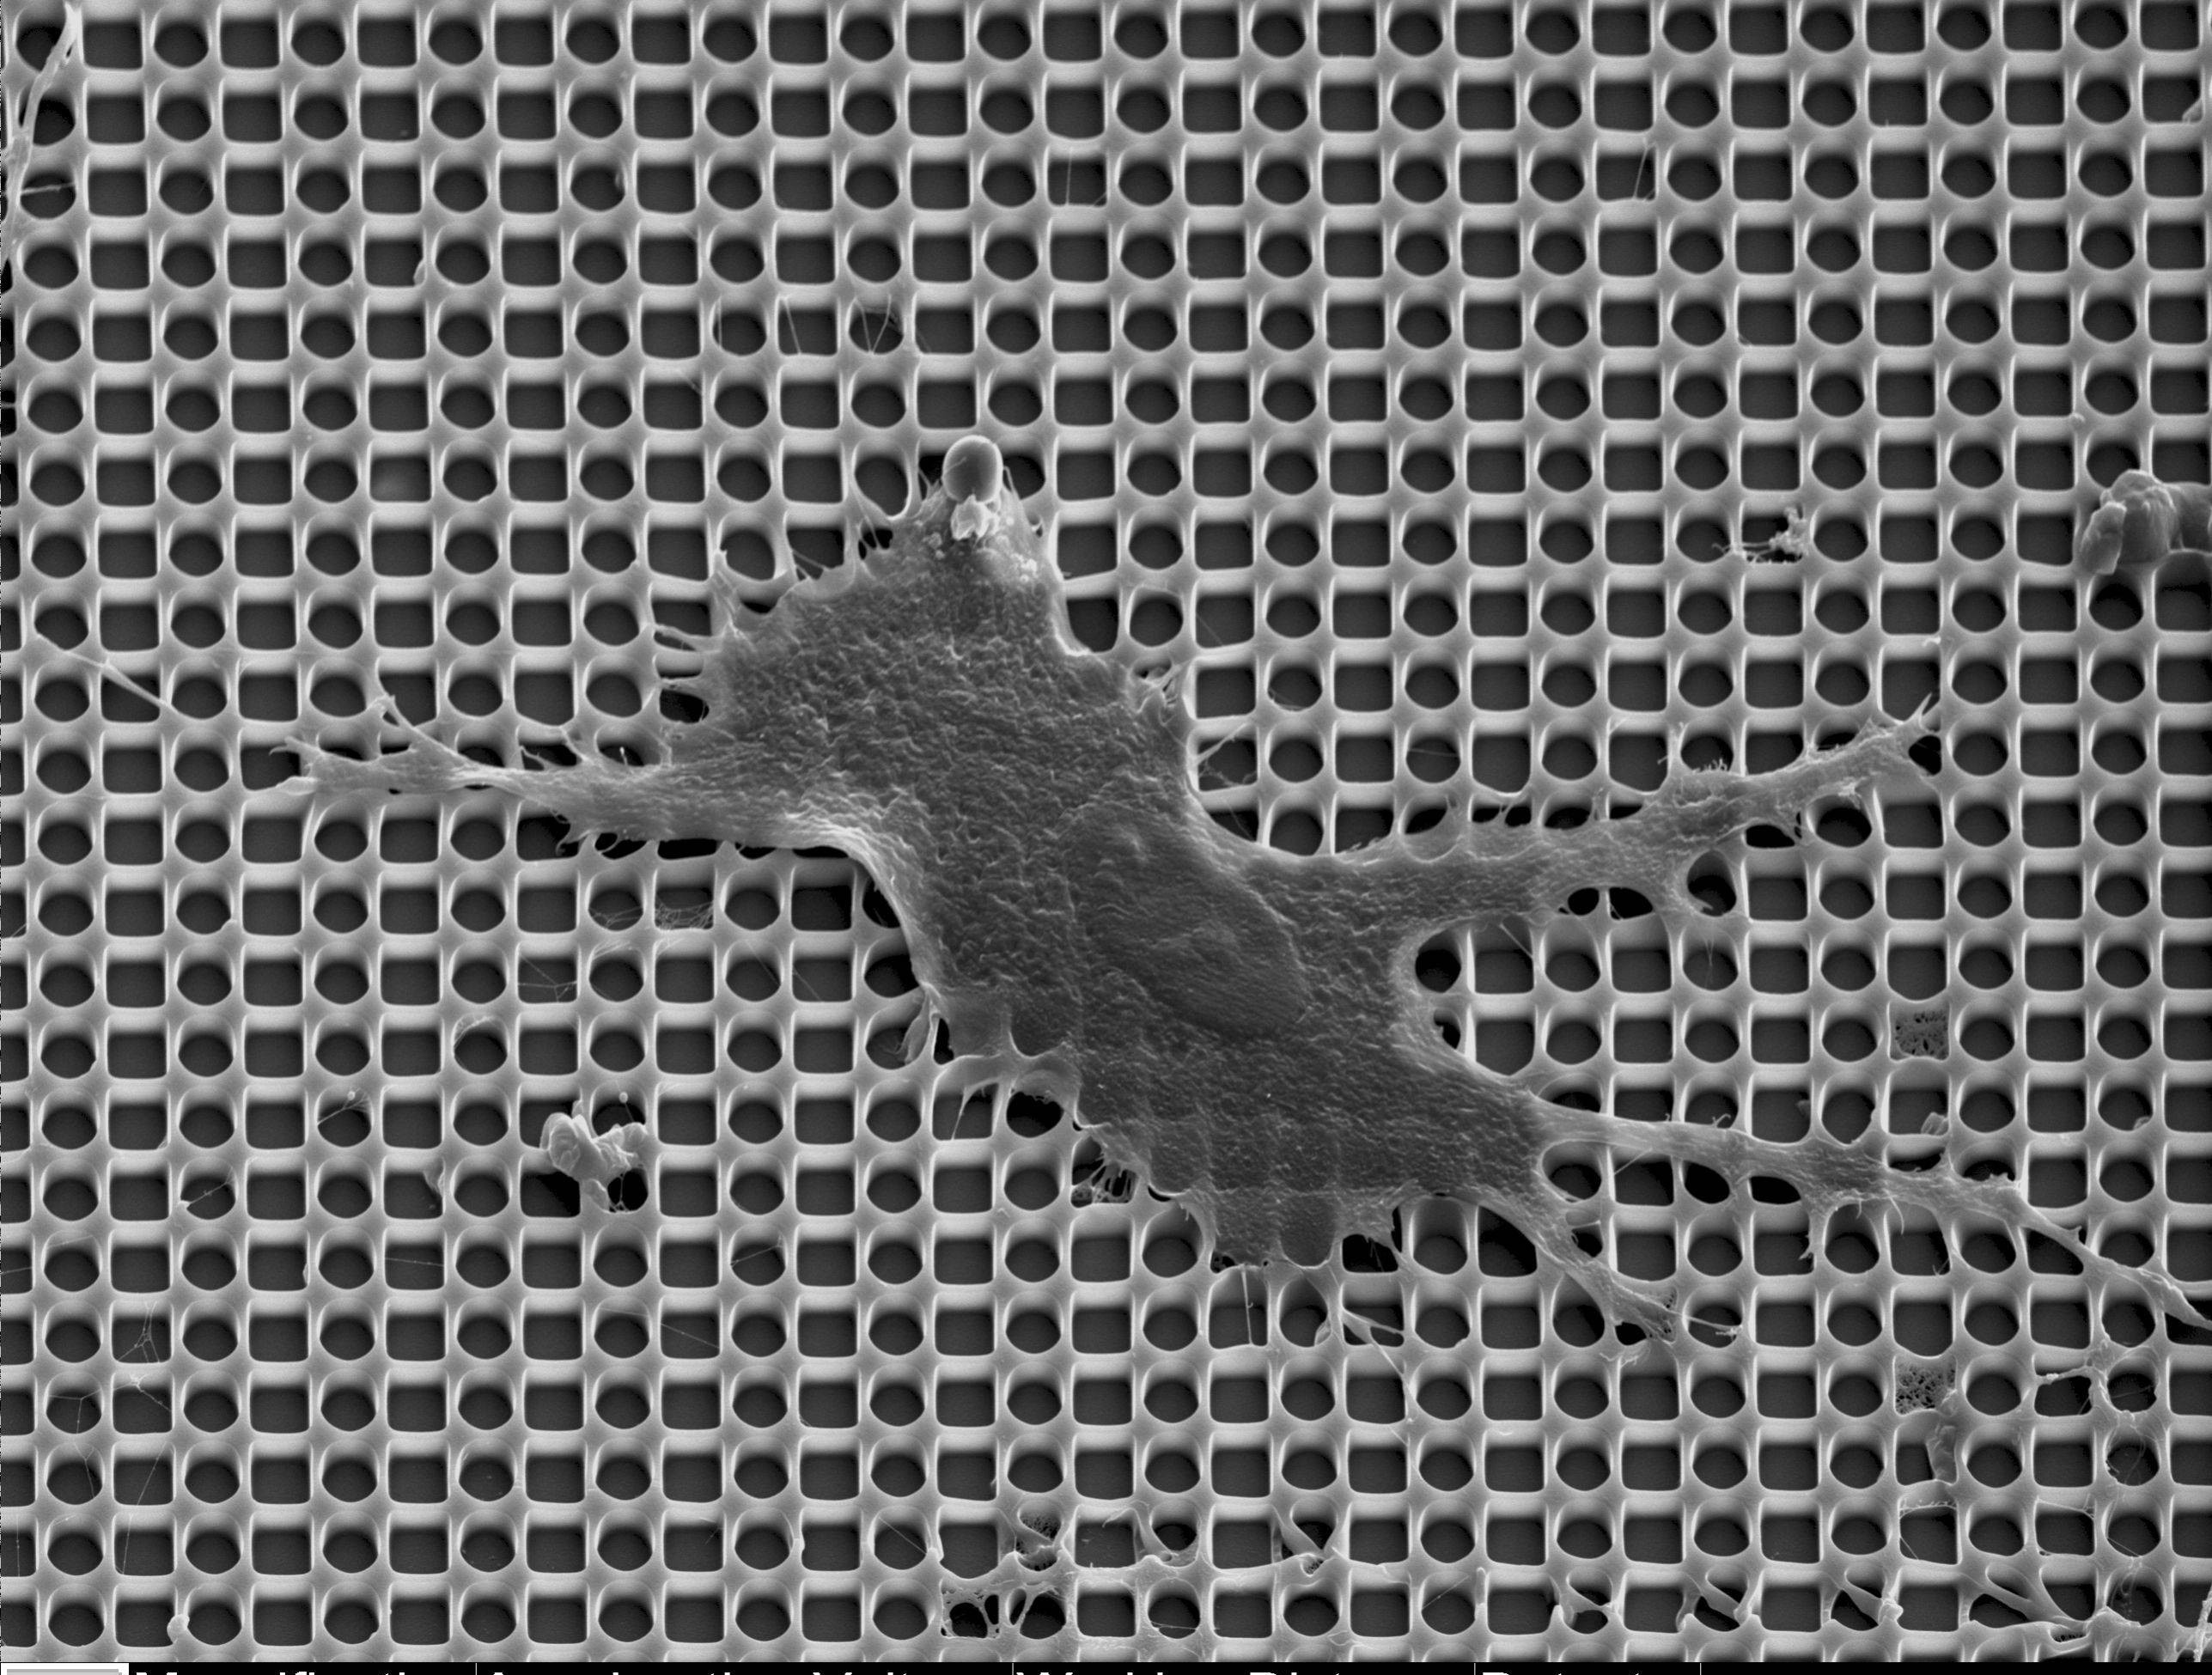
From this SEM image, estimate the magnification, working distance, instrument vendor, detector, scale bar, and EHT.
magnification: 2 K X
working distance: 12.1 mm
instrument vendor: other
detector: SE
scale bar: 20000 nm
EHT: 10 kV